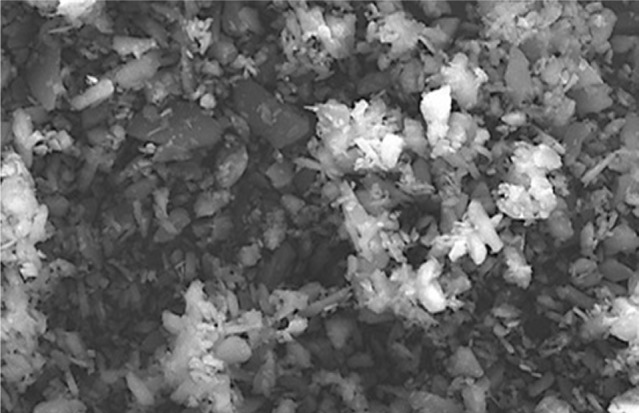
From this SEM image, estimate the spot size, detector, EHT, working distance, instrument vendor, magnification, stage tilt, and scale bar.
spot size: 2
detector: ETD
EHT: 20 kV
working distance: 10.1 mm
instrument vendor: FEI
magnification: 2 K X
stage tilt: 0°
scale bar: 20000 nm